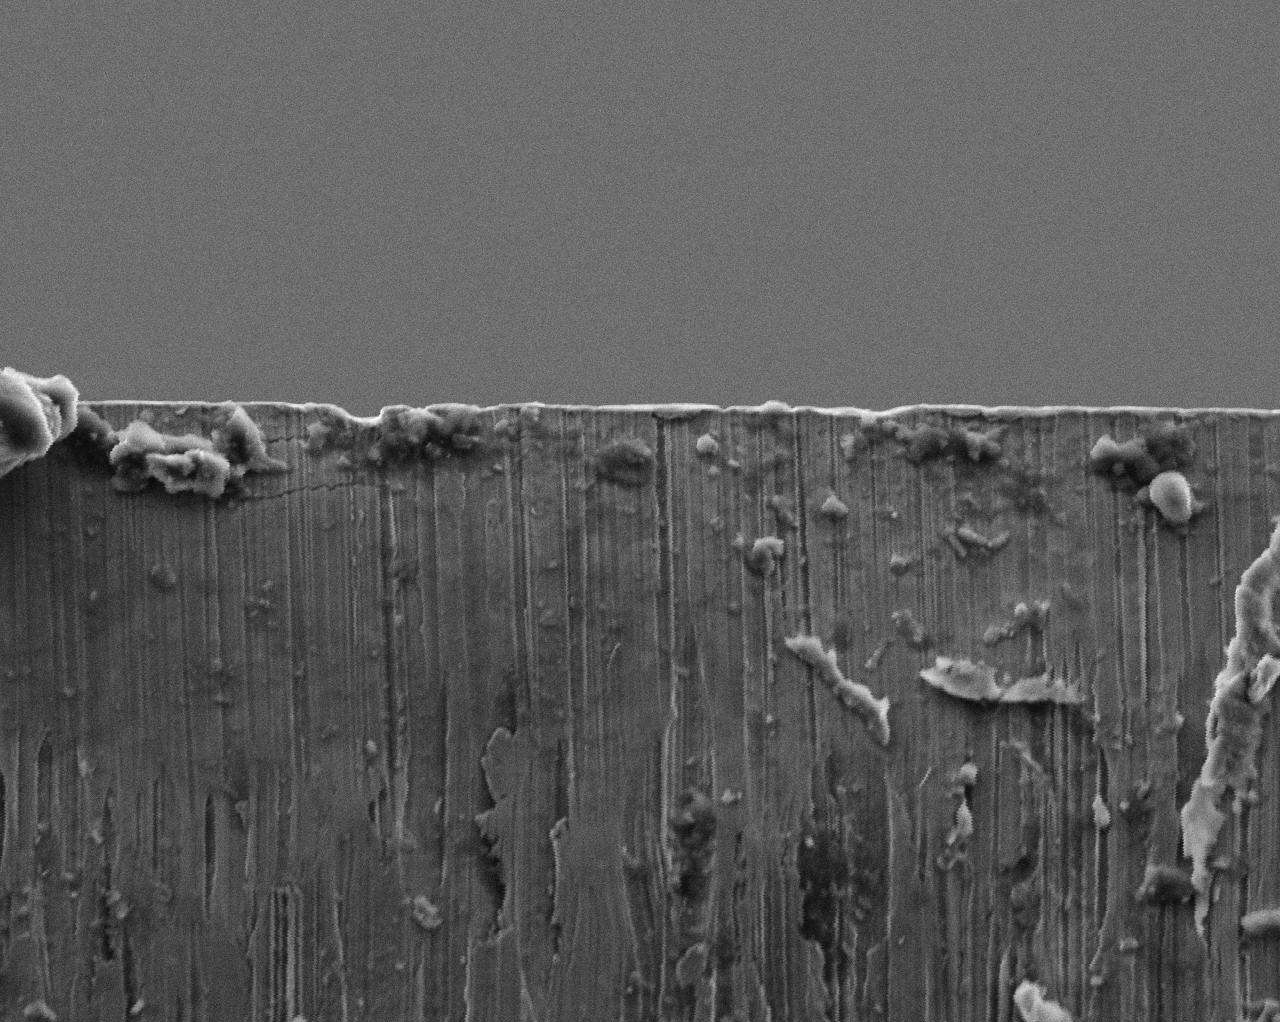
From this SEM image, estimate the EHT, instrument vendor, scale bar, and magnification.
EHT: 15 kV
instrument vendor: JEOL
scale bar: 50000 nm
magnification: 0.6 K X